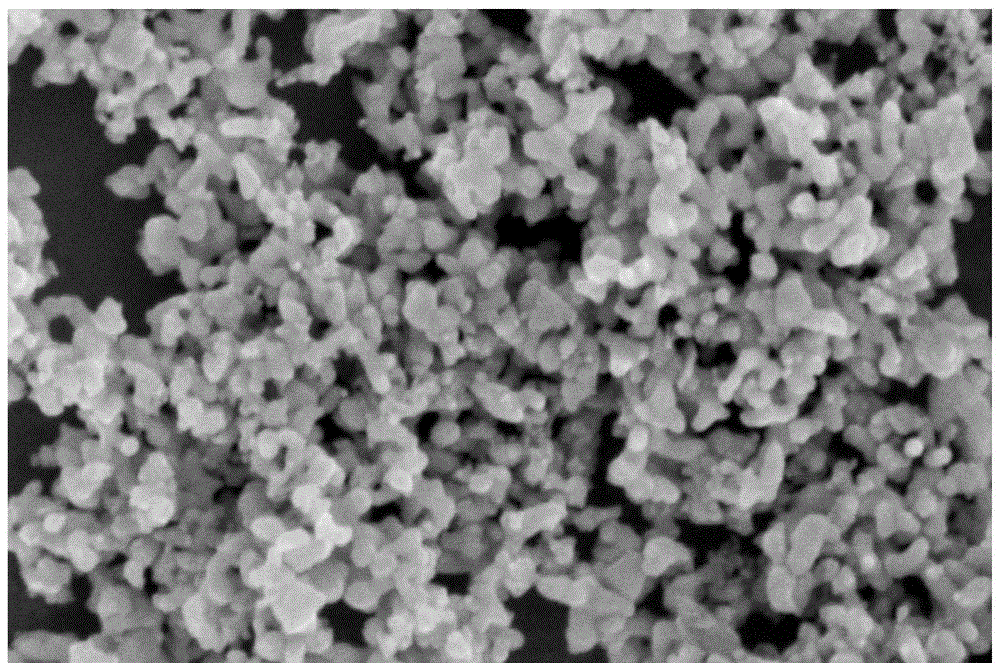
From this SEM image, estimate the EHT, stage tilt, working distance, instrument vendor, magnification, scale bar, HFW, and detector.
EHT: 3 kV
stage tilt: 0°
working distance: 2.5 mm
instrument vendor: FEI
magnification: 100 K X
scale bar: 400 nm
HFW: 1.27 µm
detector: TLD